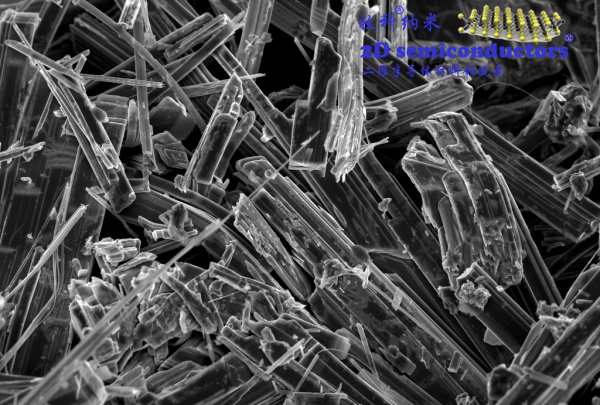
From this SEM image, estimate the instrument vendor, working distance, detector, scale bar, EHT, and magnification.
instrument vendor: Zeiss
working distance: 7 mm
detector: InLens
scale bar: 1000 nm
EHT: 5 kV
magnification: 5 K X